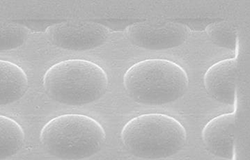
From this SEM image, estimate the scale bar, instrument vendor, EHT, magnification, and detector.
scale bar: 200000 nm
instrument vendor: JEOL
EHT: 15 kV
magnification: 0.15 K X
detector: SEI